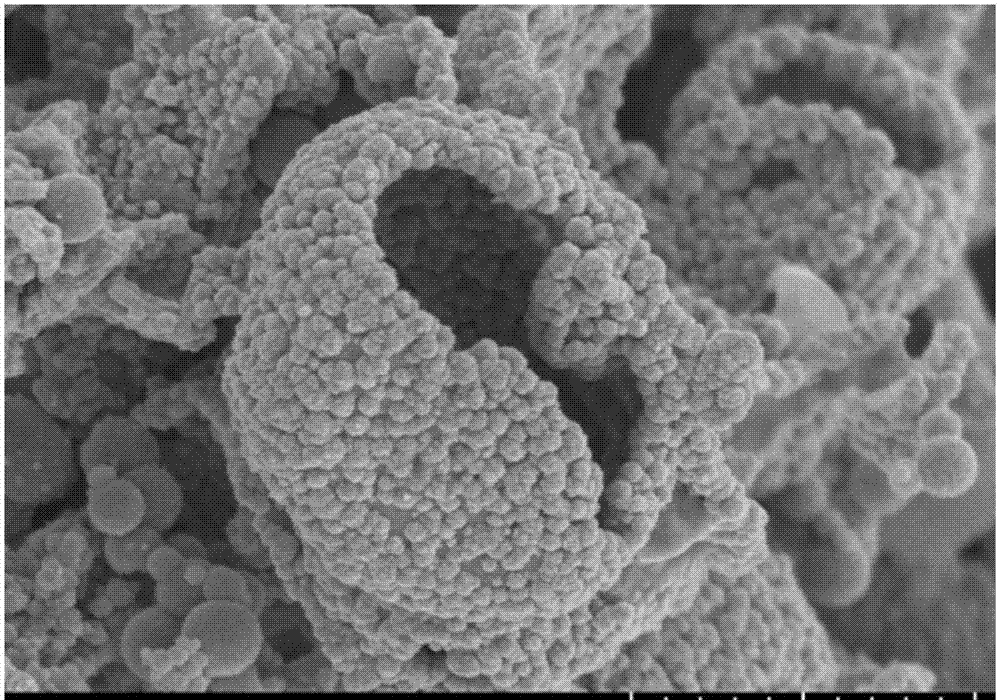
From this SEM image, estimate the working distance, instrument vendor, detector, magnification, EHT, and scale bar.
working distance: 10.3 mm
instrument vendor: Hitachi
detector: SE(UL)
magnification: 22 K X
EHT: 3 kV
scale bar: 2000 nm